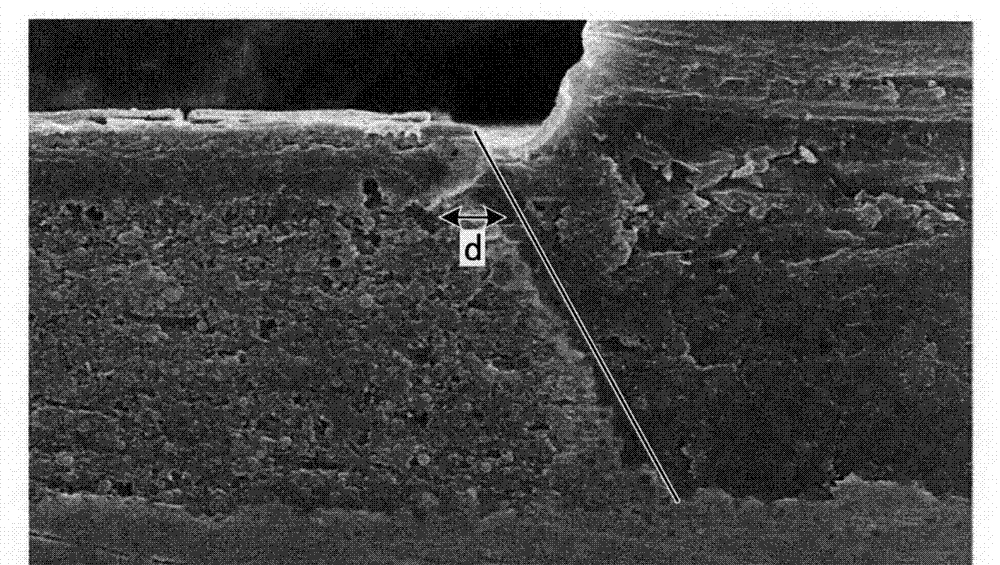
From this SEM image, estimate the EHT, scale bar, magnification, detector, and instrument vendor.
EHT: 15 kV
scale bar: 20000 nm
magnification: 2 K X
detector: SE(M)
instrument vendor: Hitachi